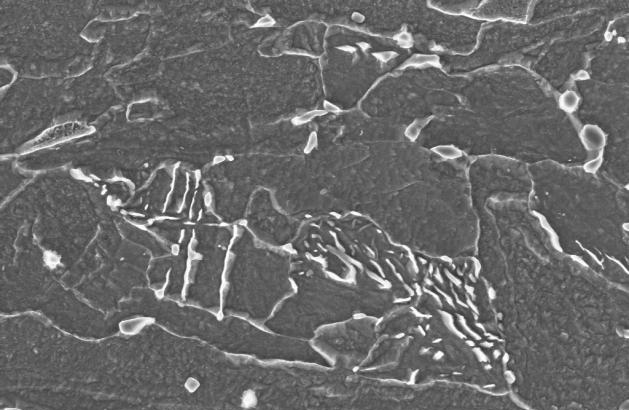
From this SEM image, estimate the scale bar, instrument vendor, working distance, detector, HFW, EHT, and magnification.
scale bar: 1000 nm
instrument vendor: Zeiss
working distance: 2.2 mm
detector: InLens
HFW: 9.932 µm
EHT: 5 kV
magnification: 30 K X